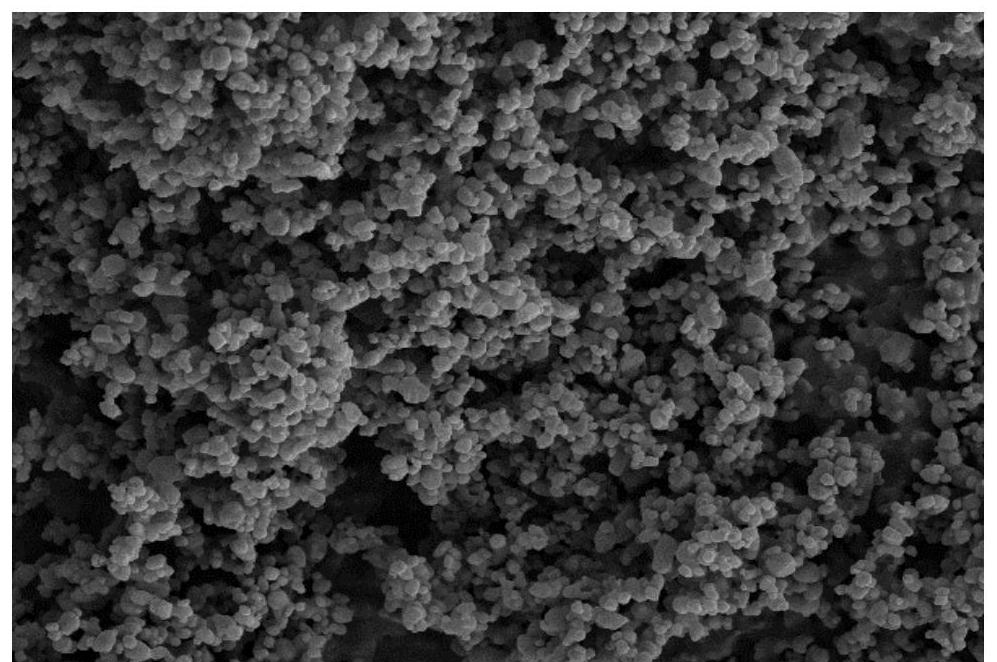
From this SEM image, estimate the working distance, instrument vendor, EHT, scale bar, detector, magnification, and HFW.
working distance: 10.08 mm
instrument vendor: other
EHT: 5 kV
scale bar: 2000 nm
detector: SE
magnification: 5 K X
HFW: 25.4 µm